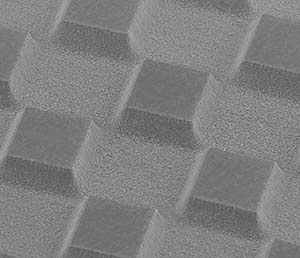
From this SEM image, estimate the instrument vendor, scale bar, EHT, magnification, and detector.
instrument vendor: JEOL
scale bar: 200000 nm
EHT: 10 kV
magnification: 0.1 K X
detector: SEI